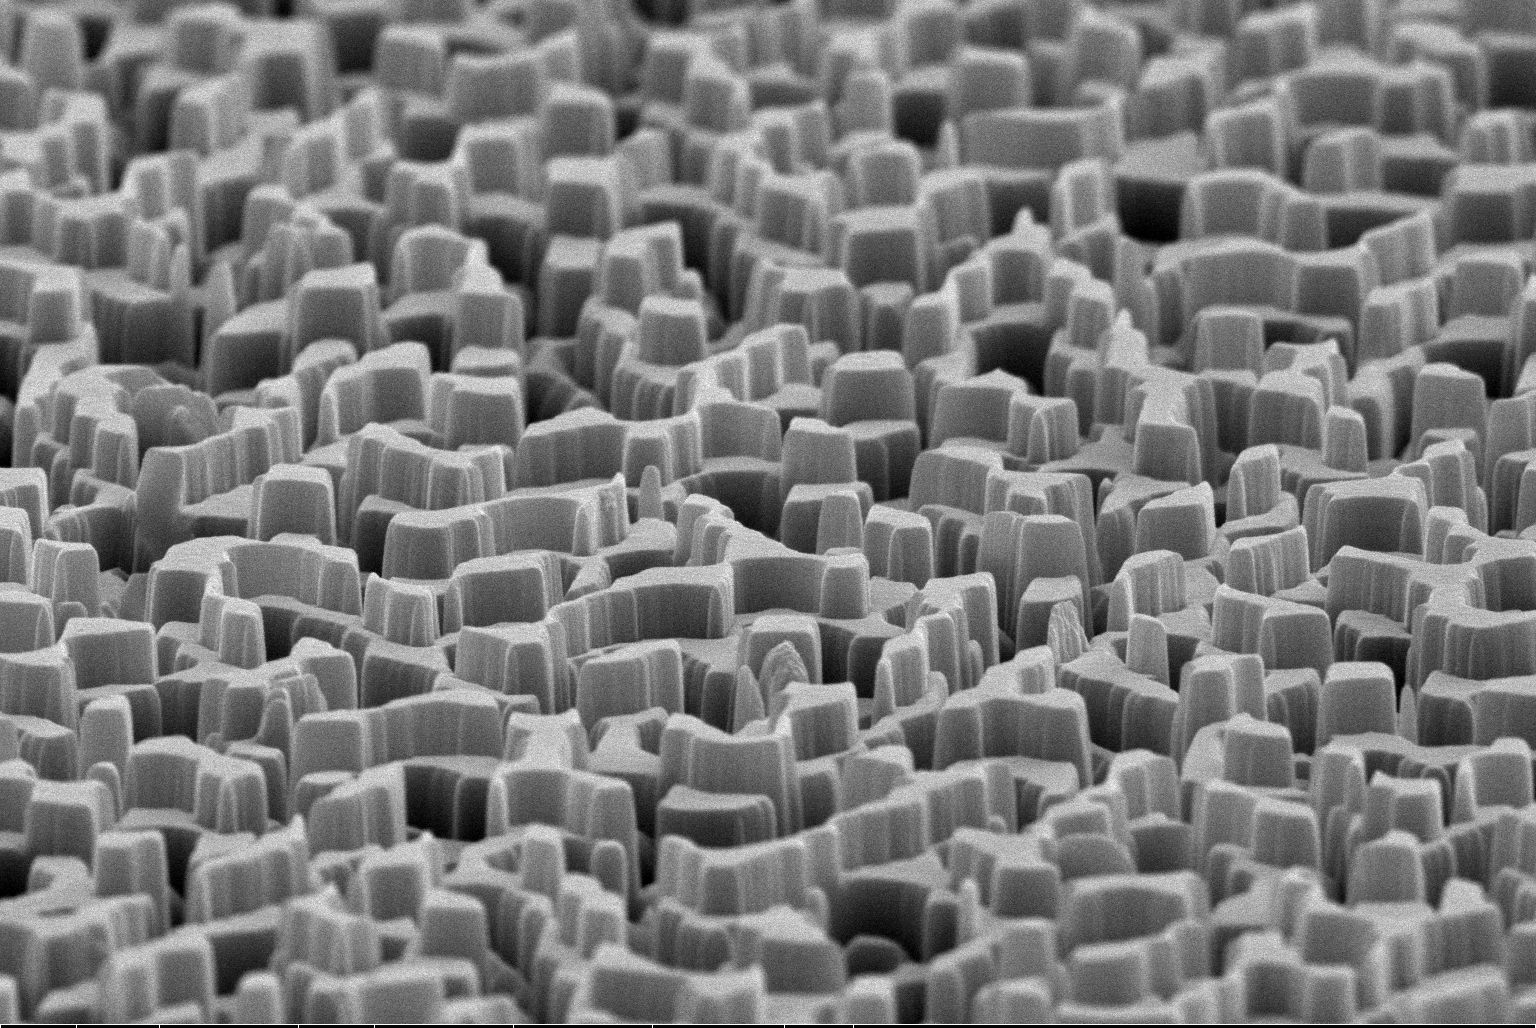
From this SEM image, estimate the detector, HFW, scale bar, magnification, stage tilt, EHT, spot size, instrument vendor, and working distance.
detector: ETD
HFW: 12.7 µm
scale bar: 5000 nm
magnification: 10 K X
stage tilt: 8°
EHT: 10 kV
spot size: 3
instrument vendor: FEI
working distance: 12.8 mm